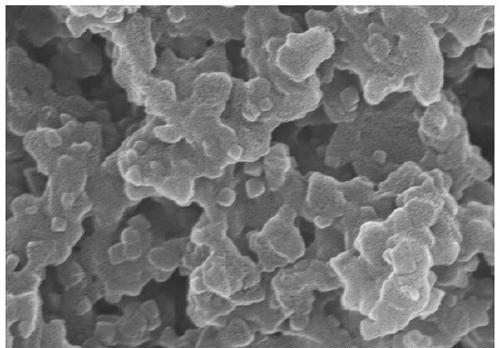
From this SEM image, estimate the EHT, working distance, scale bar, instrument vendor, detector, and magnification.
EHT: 15 kV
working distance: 7.9 mm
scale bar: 1000 nm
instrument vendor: Hitachi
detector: SE(M,LA3)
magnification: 50 K X